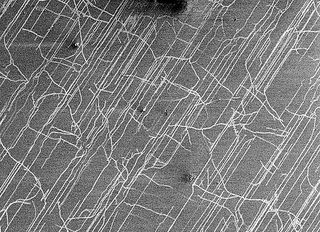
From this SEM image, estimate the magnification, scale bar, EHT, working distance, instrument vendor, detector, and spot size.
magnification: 4.256 K X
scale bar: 5000 nm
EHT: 2 kV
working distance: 4.7 mm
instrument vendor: FEI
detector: SE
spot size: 3